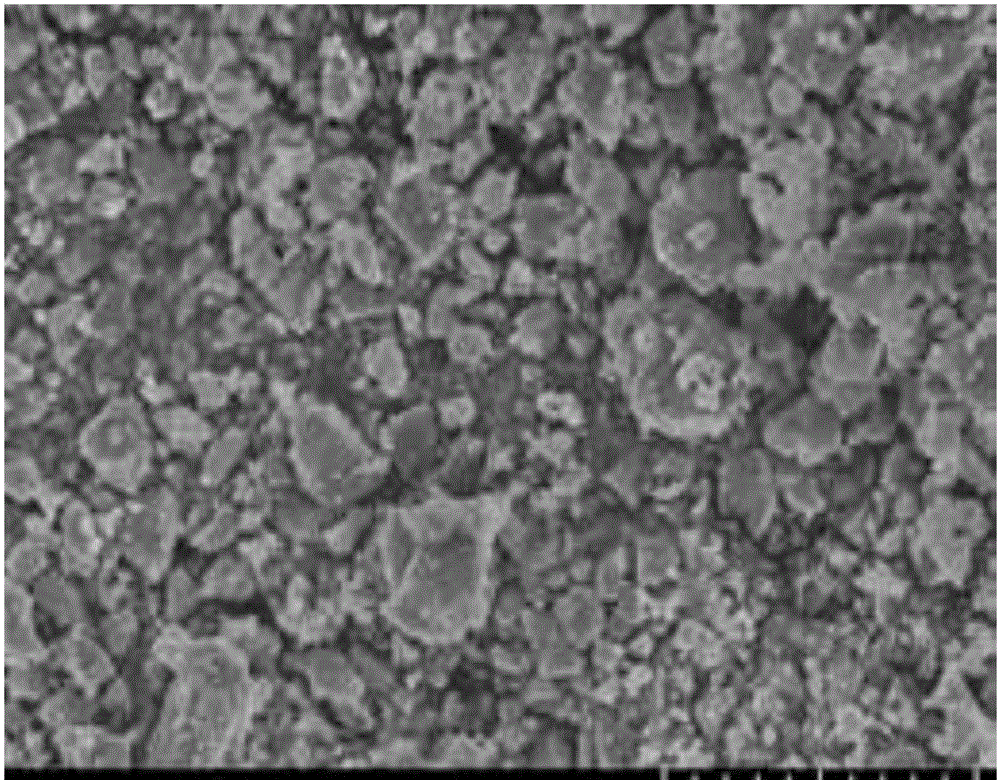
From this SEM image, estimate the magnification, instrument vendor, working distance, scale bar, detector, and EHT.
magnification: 4 K X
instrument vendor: Hitachi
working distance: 13.5 mm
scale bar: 10000 nm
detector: SE(U)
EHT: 10 kV